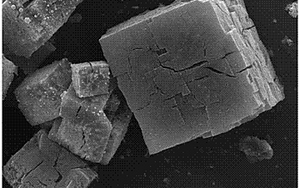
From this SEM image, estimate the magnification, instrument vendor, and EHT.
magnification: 1.4 K X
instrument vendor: Tescan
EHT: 20 kV